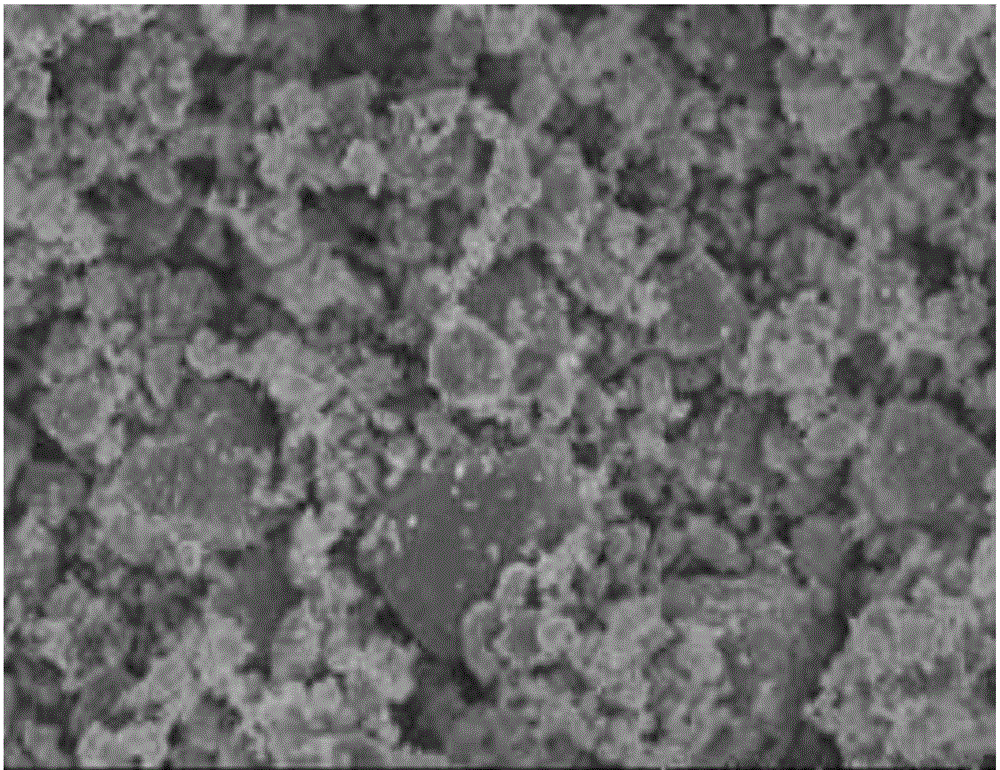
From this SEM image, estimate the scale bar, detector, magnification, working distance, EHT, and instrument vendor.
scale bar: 10000 nm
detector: SE(U)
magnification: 4 K X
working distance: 13.4 mm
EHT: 10 kV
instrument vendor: Hitachi